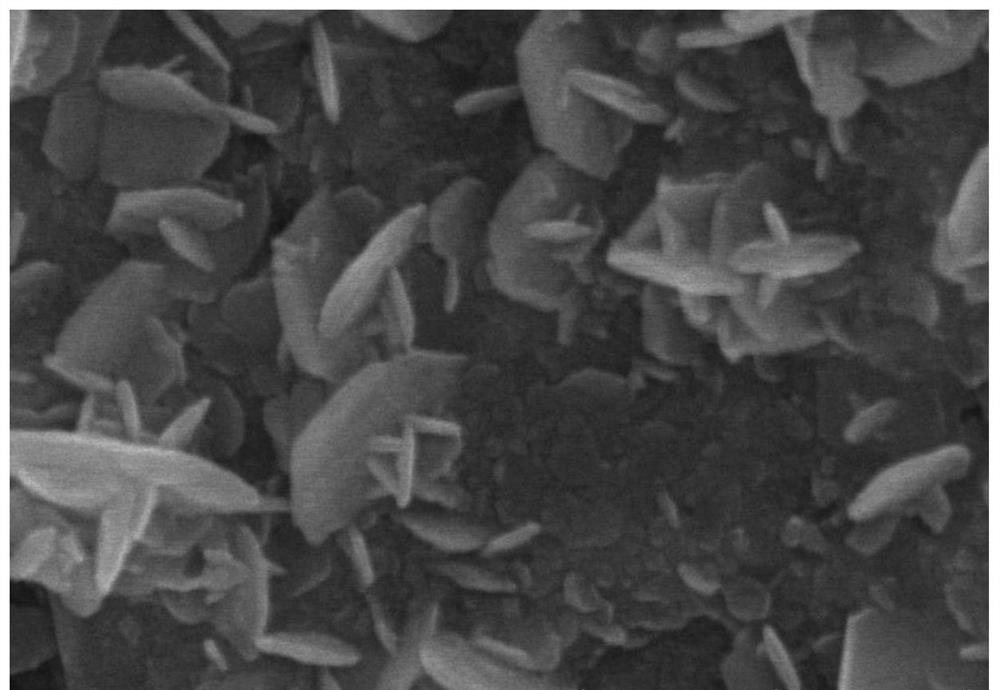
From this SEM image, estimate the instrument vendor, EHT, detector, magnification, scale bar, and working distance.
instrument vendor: Hitachi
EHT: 8 kV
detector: SE(UL)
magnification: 100 K X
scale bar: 500 nm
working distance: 8.8 mm